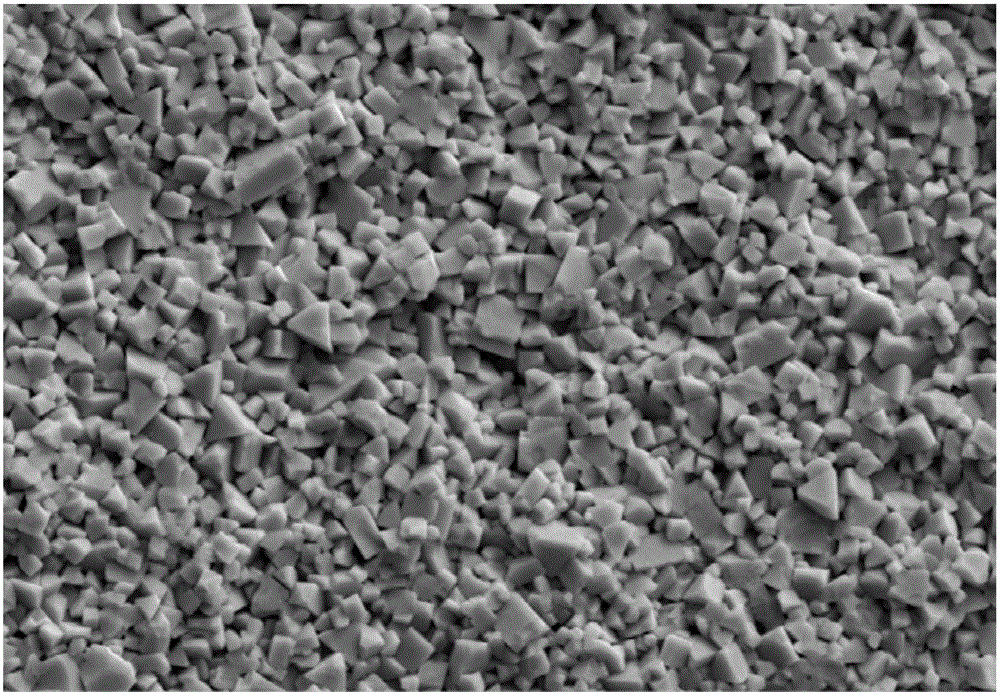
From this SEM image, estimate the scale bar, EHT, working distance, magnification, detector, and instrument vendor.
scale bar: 2000 nm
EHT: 15 kV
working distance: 10.2 mm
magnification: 5 K X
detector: SE2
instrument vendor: Zeiss